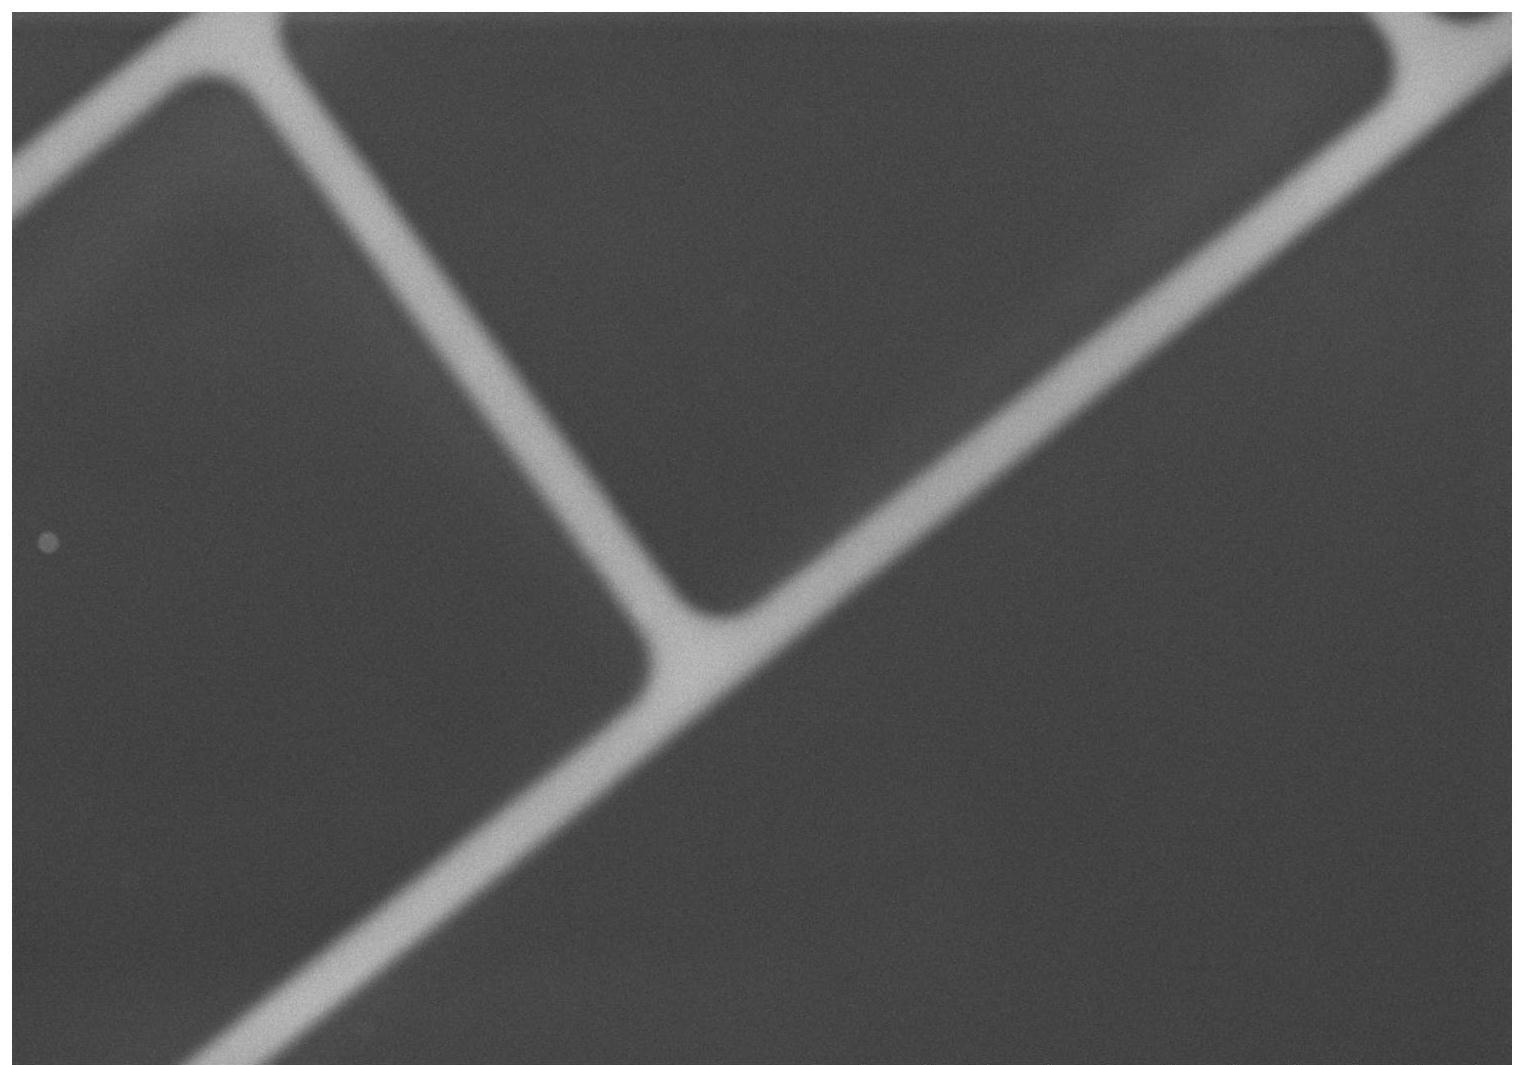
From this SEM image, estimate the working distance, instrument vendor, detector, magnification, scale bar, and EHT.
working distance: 11.4 mm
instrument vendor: Hitachi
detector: SE(L)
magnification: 0.13 K X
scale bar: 400000 nm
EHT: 15 kV